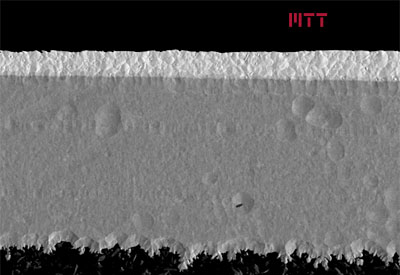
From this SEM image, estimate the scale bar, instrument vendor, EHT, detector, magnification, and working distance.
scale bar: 50000 nm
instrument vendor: Hitachi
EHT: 5 kV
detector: LA48(UL)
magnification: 1.1 K X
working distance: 8.6 mm